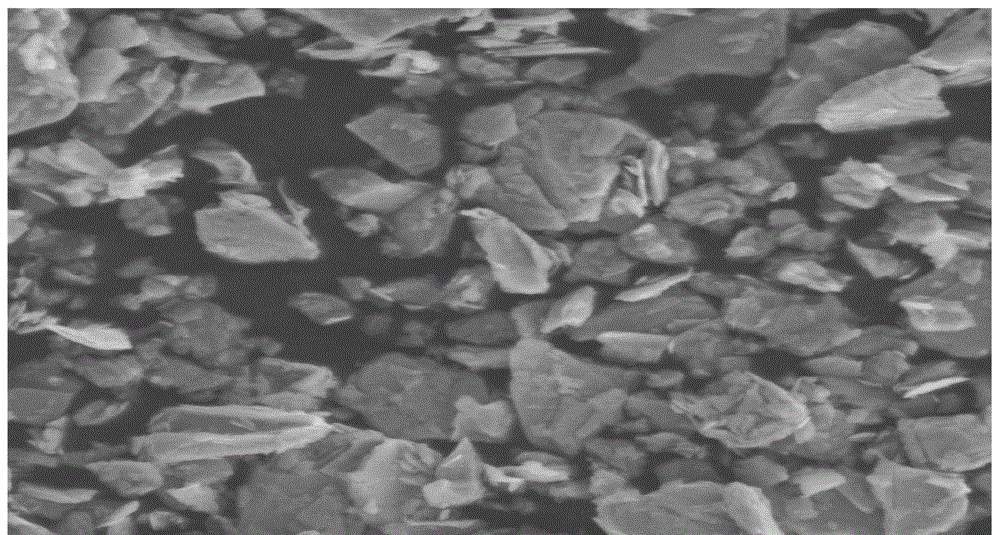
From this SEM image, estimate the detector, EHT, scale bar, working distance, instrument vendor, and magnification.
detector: LFD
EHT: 10 kV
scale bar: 10000 nm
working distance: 10.5 mm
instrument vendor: FEI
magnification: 8 K X